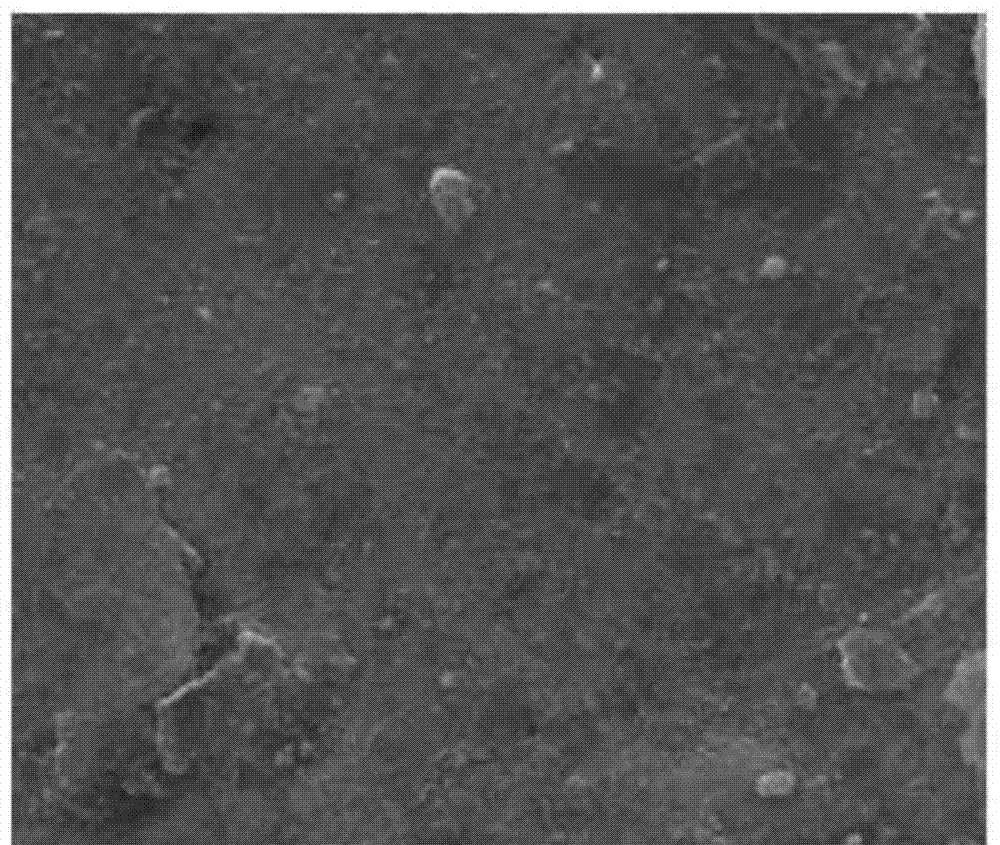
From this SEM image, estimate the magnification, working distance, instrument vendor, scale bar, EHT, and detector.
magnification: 28 K X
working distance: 8.8 mm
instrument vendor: FEI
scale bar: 4000 nm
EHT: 20 kV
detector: SE